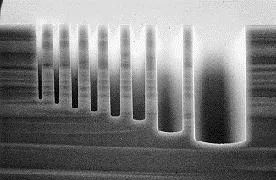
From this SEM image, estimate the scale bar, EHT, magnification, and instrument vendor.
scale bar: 50000 nm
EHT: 20 kV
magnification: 1 K X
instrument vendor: Hitachi